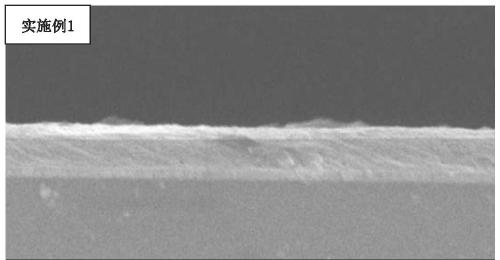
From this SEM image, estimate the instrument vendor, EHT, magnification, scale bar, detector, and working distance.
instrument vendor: JEOL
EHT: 5 kV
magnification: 10 K X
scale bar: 1000 nm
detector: SEI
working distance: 9.3 mm